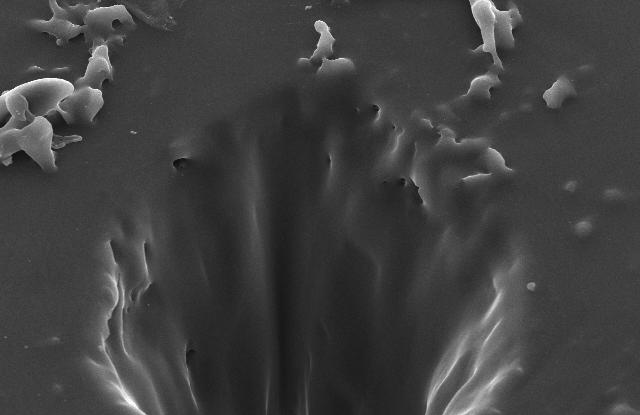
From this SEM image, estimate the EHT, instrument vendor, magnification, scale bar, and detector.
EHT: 20 kV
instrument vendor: JEOL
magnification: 0.7 K X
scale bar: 20000 nm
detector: SEI